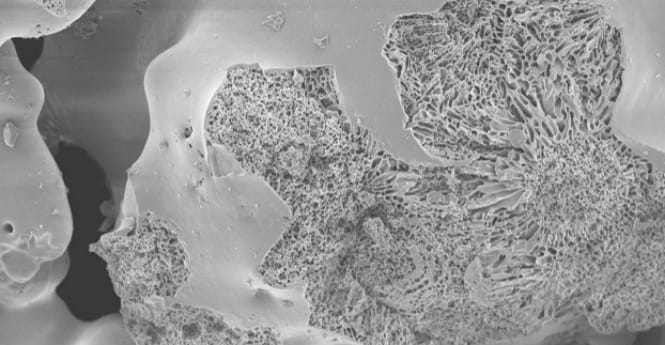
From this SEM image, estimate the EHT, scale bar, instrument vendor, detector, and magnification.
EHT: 5 kV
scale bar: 50000 nm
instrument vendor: JEOL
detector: SED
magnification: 0.4 K X